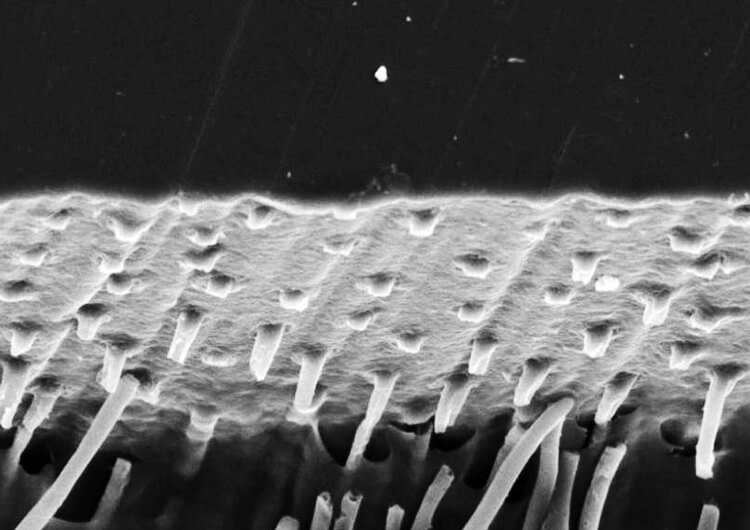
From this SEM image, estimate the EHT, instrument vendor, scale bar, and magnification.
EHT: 10 kV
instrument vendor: JEOL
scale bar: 10000 nm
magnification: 2 K X